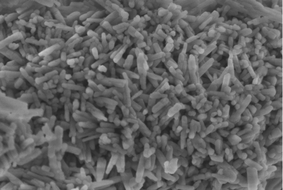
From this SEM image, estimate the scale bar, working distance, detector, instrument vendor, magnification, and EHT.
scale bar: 1000 nm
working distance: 6.4 mm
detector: SE+BSE(U)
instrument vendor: Hitachi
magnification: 50 K X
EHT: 2 kV